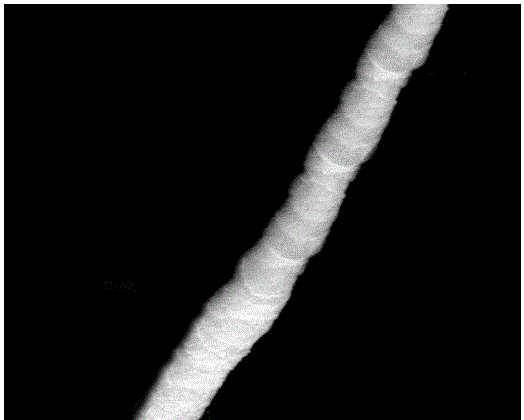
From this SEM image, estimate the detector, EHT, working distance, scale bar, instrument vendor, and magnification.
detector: LFD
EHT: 10 kV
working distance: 11.3 mm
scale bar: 1000 nm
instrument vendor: FEI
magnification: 150 K X